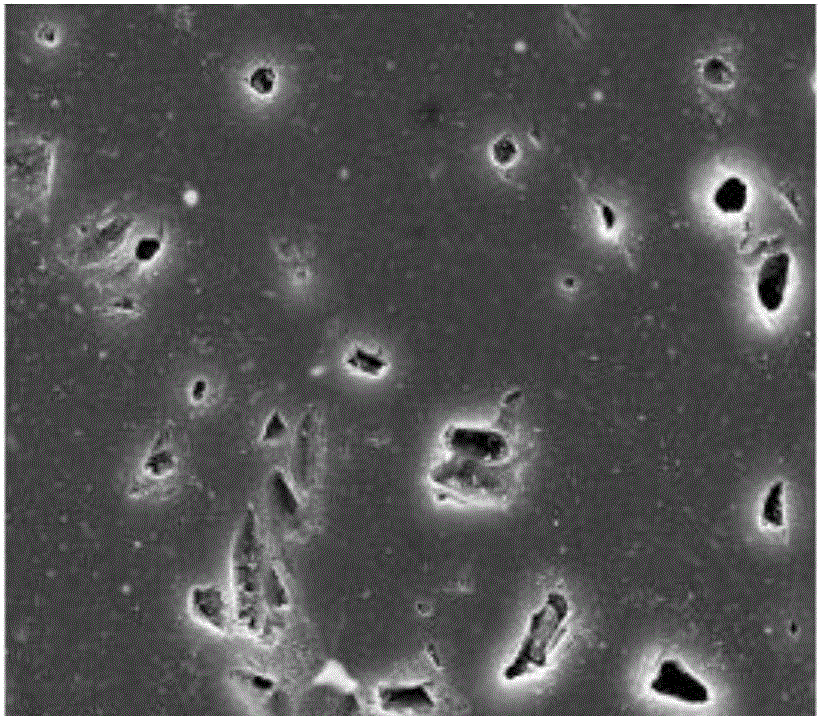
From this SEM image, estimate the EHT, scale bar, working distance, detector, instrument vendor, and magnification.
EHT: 20 kV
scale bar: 20000 nm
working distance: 9.9 mm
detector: SE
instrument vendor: FEI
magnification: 5 K X